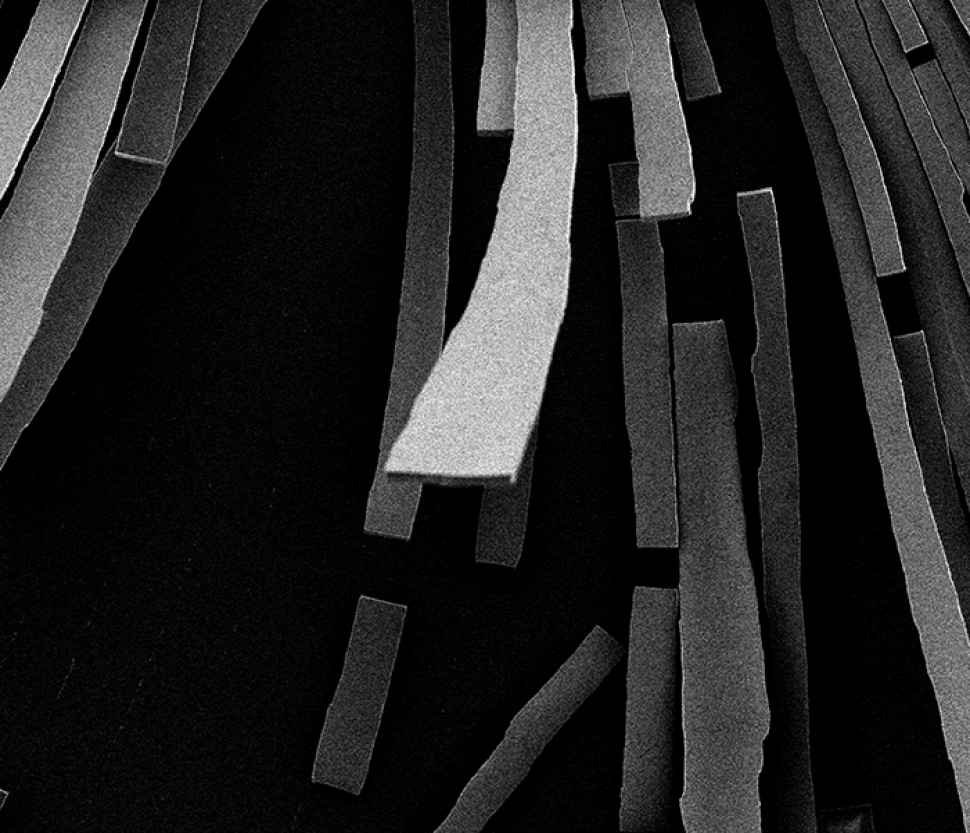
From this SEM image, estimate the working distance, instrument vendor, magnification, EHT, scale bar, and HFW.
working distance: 6.6 mm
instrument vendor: FEI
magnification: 0.589 K X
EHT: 5 kV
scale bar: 200000 nm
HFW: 506 µm